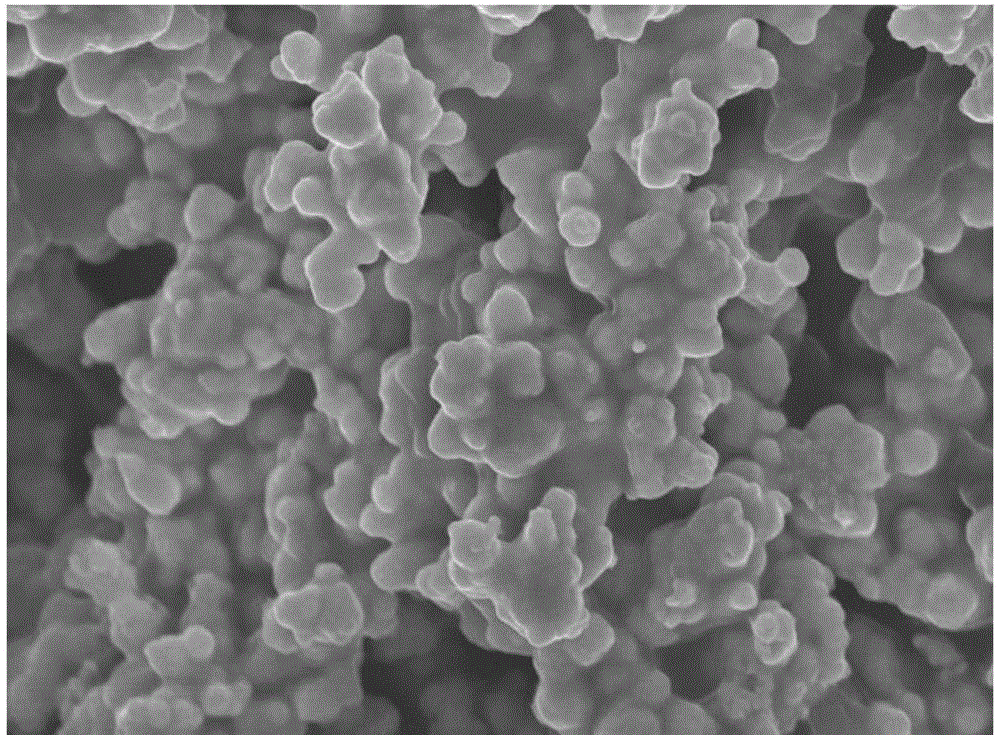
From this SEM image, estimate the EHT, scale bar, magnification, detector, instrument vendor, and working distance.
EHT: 15 kV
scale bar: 1000 nm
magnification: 10 K X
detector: SEI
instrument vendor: JEOL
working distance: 9.2 mm